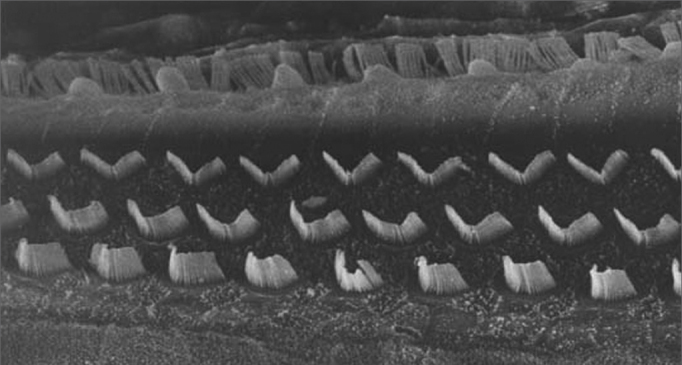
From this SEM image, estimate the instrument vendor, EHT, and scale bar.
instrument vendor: JEOL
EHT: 15 kV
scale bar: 10000 nm